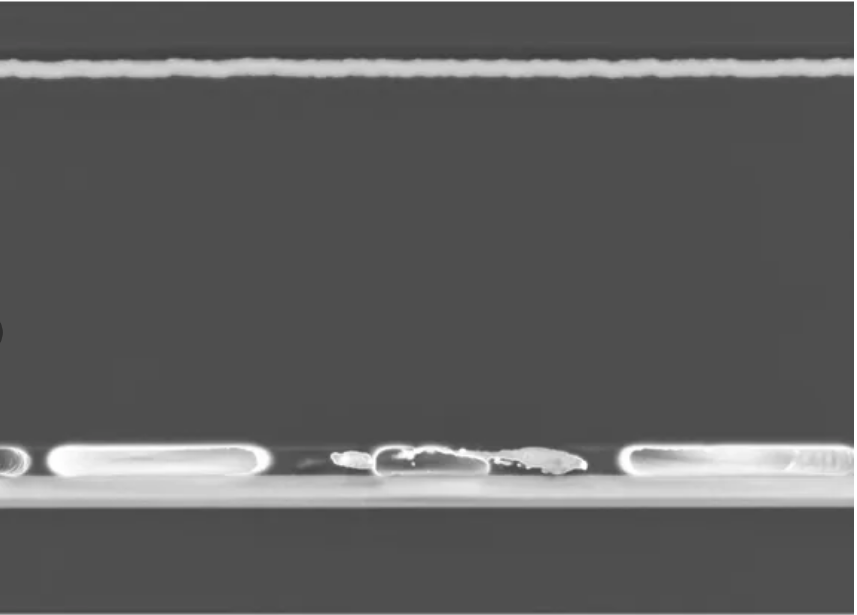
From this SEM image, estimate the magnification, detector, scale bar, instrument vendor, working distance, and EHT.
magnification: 6.64 K X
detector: InLens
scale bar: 1000 nm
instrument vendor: Zeiss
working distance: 4.4 mm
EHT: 3 kV